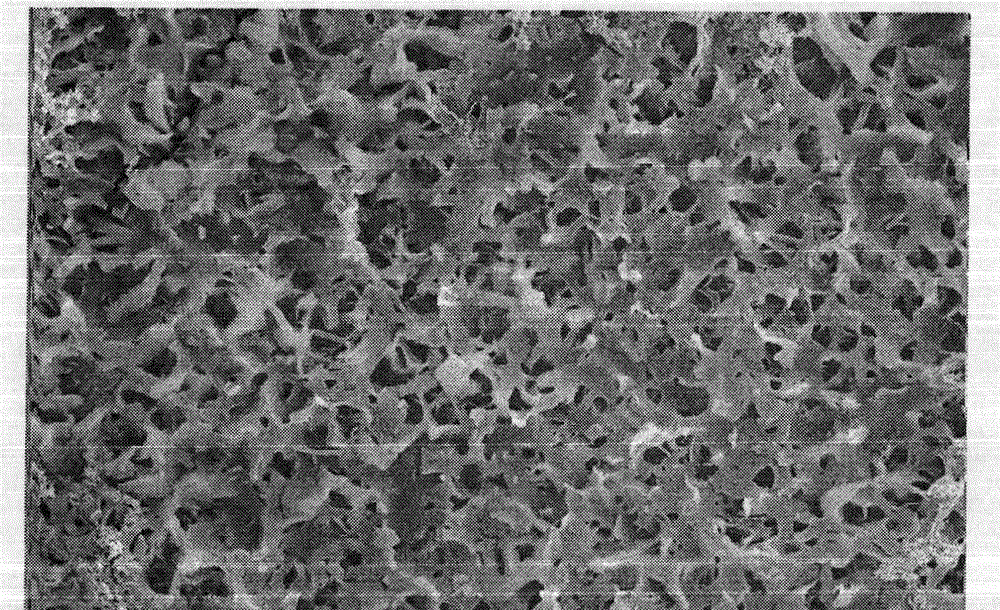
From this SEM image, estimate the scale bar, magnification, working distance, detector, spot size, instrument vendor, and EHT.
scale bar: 5000 nm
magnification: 10 K X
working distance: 9.1 mm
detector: SE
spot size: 3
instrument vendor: FEI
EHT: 20 kV